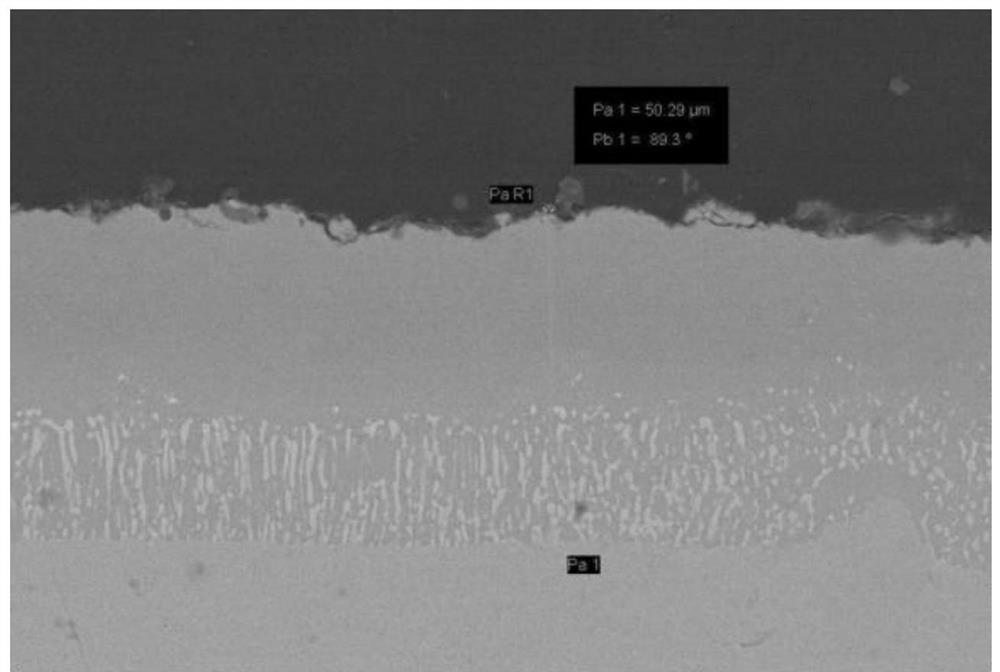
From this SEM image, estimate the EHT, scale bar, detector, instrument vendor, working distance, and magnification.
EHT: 20 kV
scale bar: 10000 nm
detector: AsB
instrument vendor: Zeiss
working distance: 18.9 mm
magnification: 2 K X